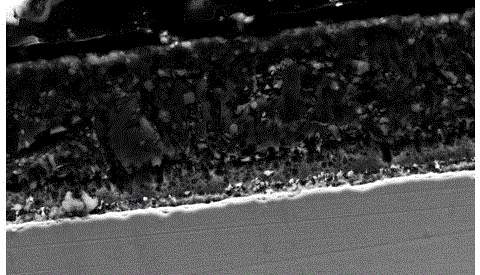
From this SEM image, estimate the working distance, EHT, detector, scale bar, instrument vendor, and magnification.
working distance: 10.5 mm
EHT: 20 kV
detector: SE2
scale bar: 10000 nm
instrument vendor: Zeiss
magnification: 1.46 K X